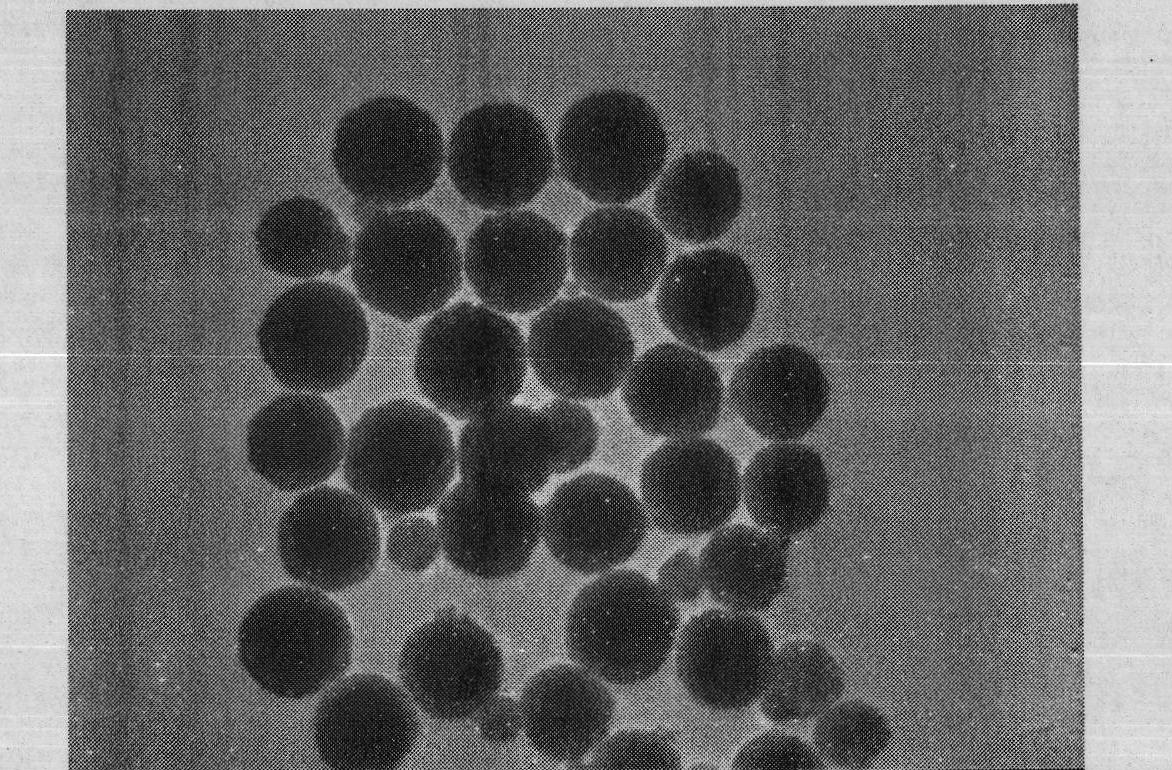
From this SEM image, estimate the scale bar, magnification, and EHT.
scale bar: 600 nm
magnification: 30 K X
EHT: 200 kV